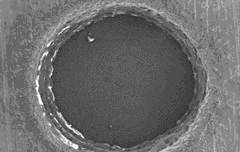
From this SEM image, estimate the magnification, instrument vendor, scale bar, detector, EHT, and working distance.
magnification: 0.5 K X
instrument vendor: Hitachi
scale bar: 100000 nm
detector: SE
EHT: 10 kV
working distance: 19.1 mm